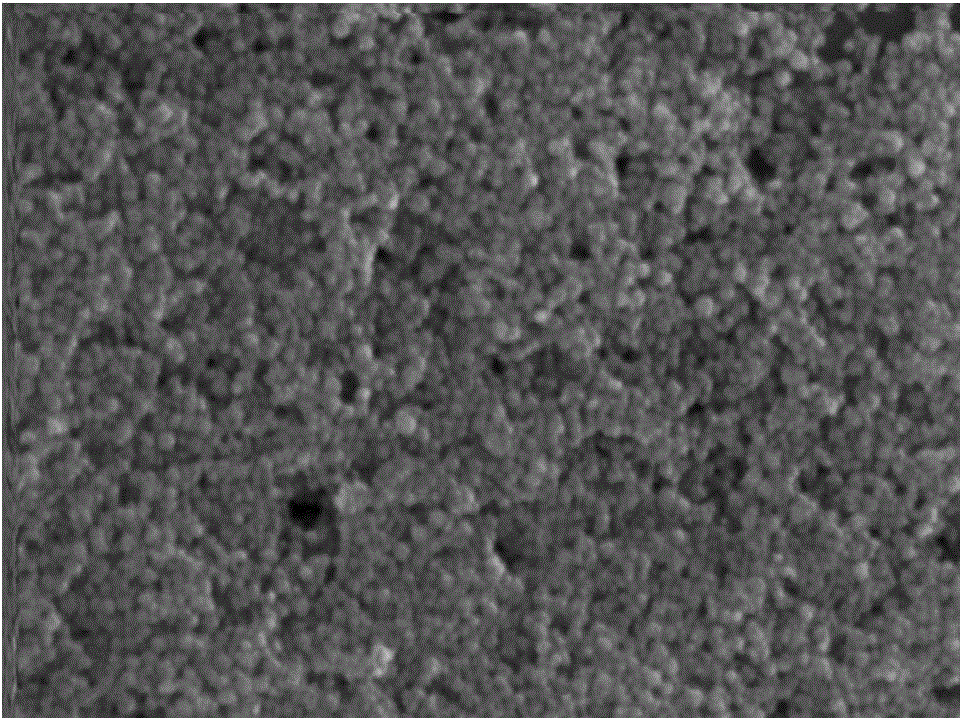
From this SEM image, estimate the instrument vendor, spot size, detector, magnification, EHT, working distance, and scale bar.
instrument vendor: FEI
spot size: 5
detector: TLD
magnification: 40 K X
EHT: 10 kV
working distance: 3.8 mm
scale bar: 500 nm